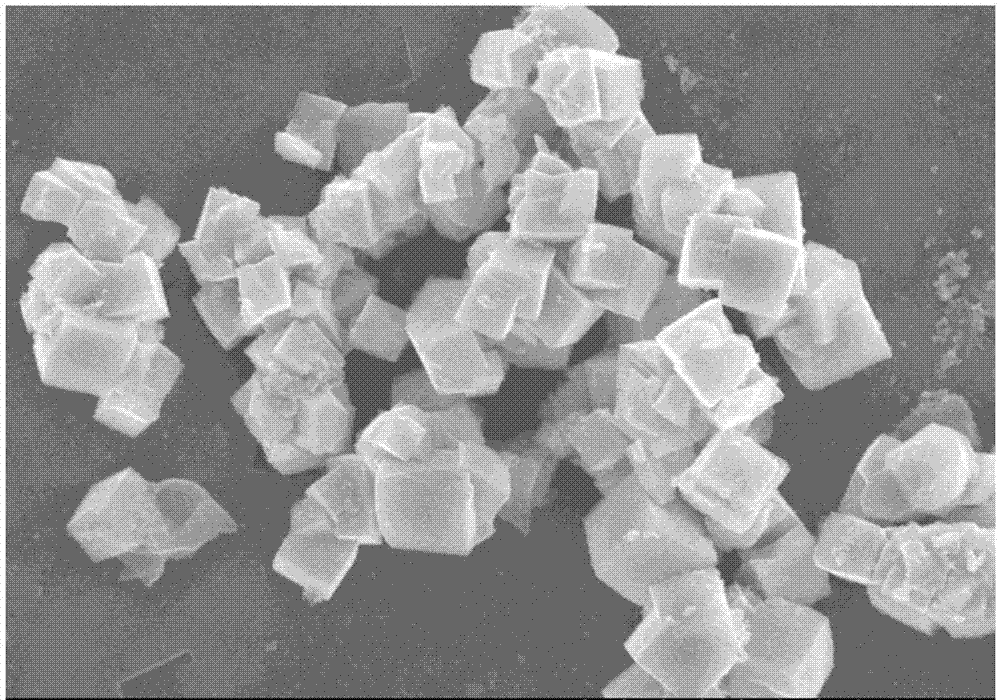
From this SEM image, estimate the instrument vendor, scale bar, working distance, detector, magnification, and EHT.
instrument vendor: Hitachi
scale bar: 10000 nm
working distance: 9.8 mm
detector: SE(M)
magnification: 5 K X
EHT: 20 kV